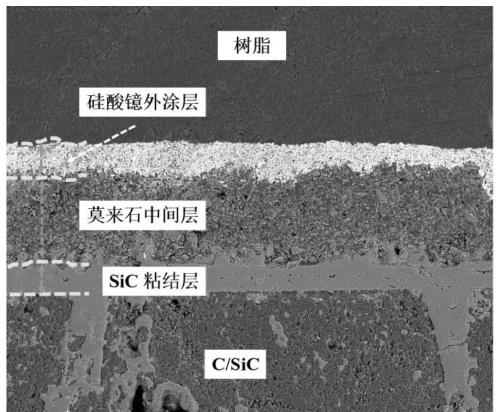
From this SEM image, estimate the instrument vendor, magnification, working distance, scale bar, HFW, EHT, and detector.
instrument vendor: FEI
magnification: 0.4 K X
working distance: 5.2 mm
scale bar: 200000 nm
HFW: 750 µm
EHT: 18 kV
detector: BSED